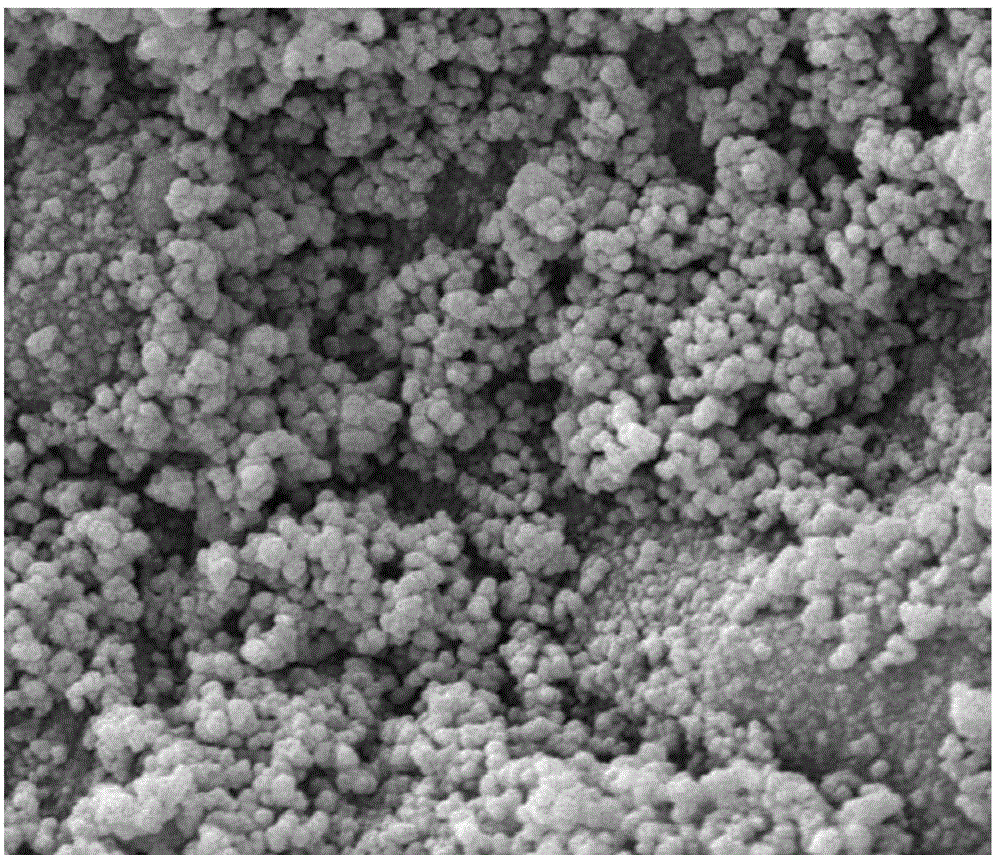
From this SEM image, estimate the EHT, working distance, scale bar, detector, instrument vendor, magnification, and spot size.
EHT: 20 kV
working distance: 7 mm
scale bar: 5000 nm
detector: ETD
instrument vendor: FEI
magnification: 20 K X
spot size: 3